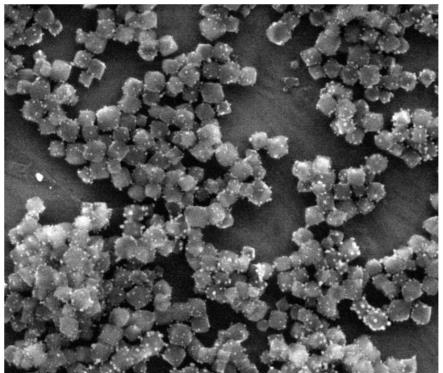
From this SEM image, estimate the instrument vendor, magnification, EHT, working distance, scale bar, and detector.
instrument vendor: FEI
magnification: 100 K X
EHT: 20 kV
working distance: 9.2 mm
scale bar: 1000 nm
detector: ETD